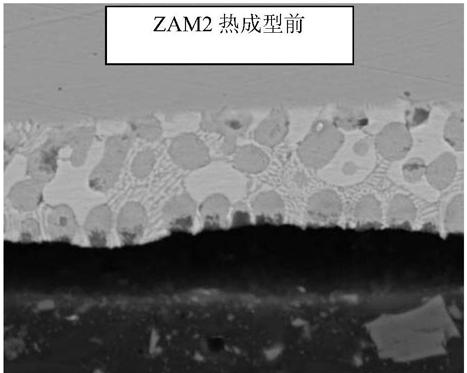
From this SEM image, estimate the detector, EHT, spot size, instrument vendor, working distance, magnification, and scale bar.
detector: SSD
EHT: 20 kV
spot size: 4.5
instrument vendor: FEI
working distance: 8 mm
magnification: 2 K X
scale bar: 20000 nm